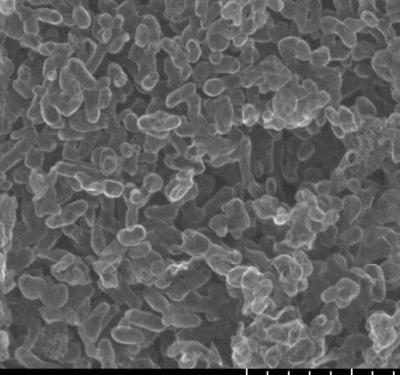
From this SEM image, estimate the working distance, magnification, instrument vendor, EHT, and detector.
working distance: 4.3 mm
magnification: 100 K X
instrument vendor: Hitachi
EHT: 15 kV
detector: SE(U)